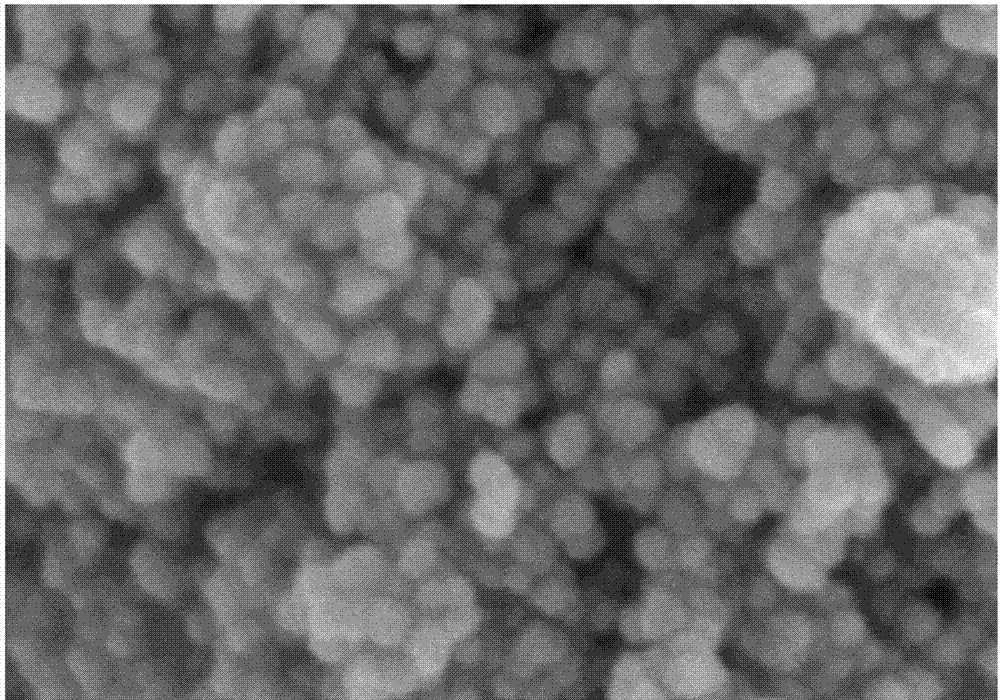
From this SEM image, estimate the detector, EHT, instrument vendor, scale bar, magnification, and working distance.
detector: SE(M)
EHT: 3 kV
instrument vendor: Hitachi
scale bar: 200 nm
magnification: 200 K X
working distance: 9.6 mm